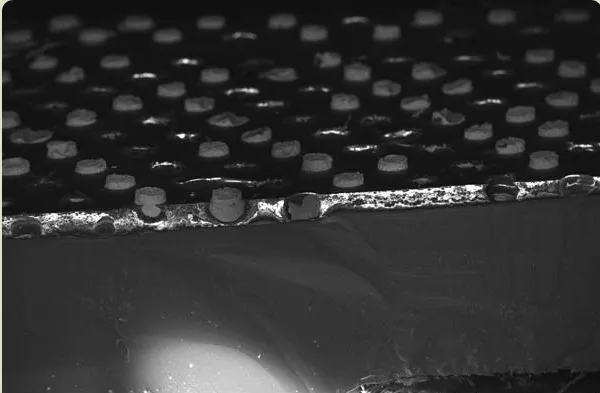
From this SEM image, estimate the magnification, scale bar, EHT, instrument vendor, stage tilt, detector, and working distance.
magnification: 0.113 K X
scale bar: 200000 nm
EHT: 15 kV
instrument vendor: Zeiss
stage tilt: -15°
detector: InLens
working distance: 4 mm